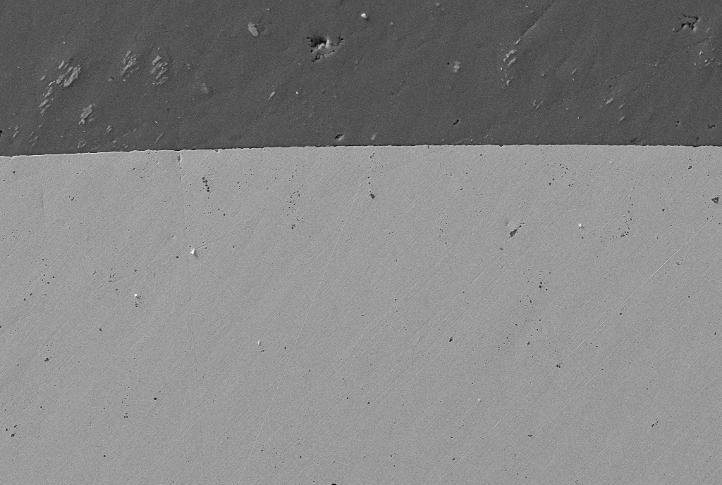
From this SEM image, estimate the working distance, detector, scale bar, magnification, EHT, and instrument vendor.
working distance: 7 mm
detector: SE2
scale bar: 20000 nm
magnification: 1 K X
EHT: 15 kV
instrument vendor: Zeiss